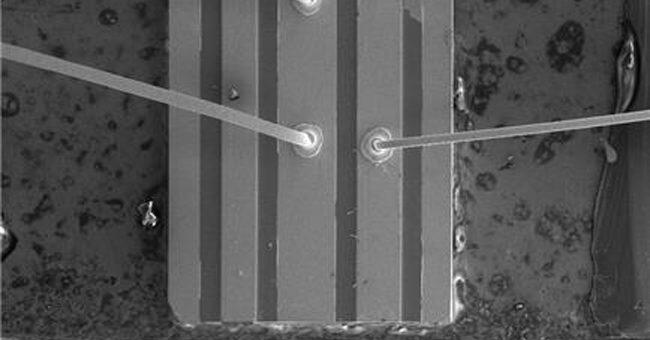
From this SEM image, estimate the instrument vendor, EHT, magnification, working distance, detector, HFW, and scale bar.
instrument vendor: FEI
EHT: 15 kV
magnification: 0.07 K X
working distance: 8.3 mm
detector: ETD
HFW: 1830 µm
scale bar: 500000 nm